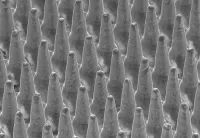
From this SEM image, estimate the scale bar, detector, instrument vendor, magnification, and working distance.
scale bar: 300000 nm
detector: SE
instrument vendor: Hitachi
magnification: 0.15 K X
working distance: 25.1 mm